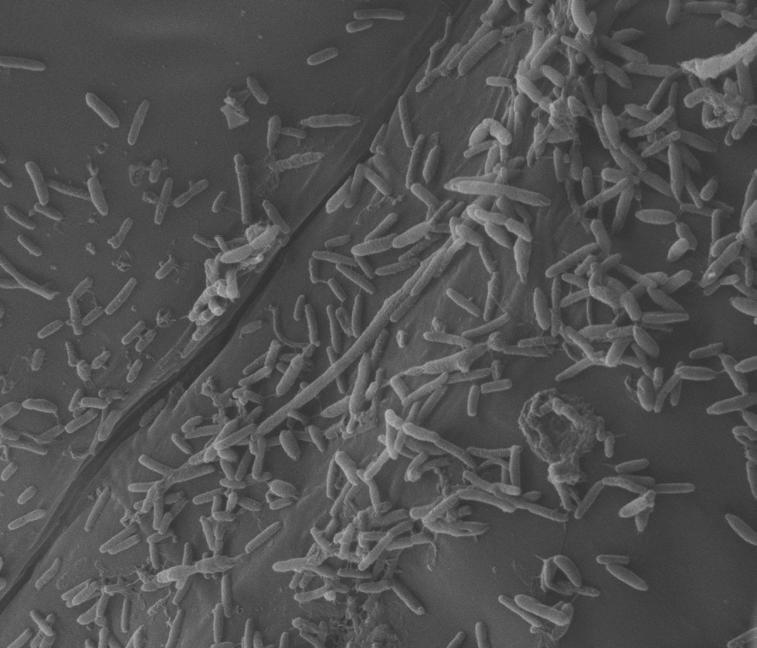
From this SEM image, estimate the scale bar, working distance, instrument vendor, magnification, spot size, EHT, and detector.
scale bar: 10000 nm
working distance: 9.9 mm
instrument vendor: FEI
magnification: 10 K X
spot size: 2.5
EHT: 20 kV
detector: ETD